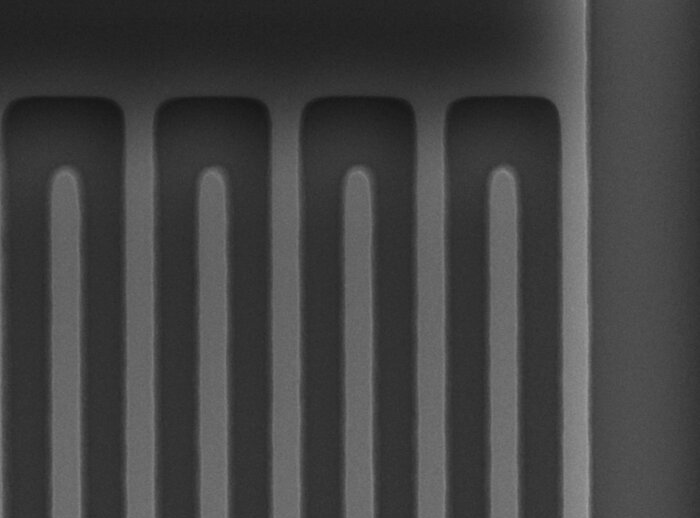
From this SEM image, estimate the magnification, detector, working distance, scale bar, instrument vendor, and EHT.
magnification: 10 K X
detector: LED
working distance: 10 mm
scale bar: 1000 nm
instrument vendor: JEOL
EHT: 10 kV